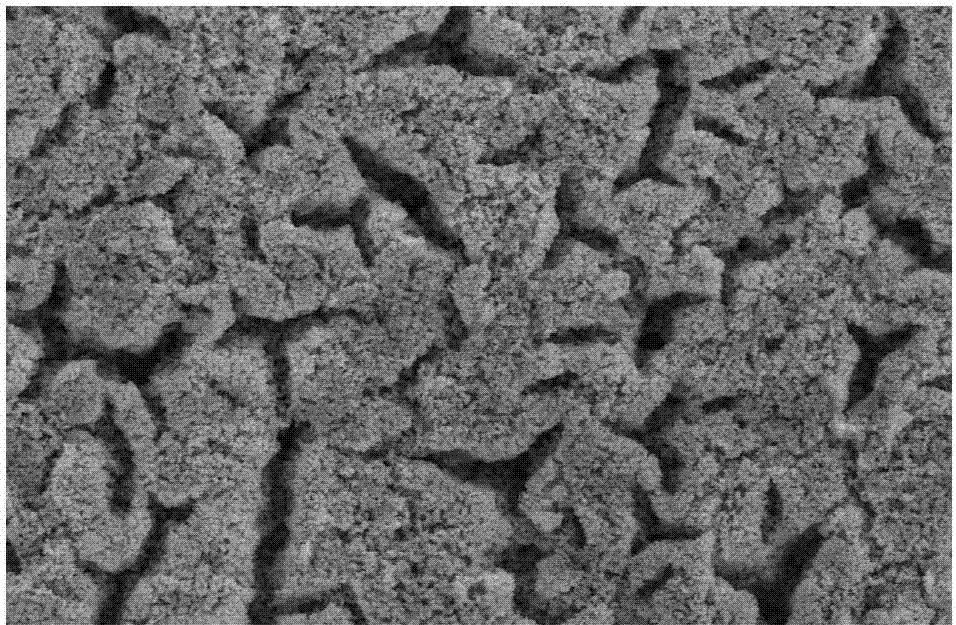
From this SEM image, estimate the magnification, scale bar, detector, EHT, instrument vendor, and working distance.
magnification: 5 K X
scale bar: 10000 nm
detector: SE(M)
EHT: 15 kV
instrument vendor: Hitachi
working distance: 12.7 mm